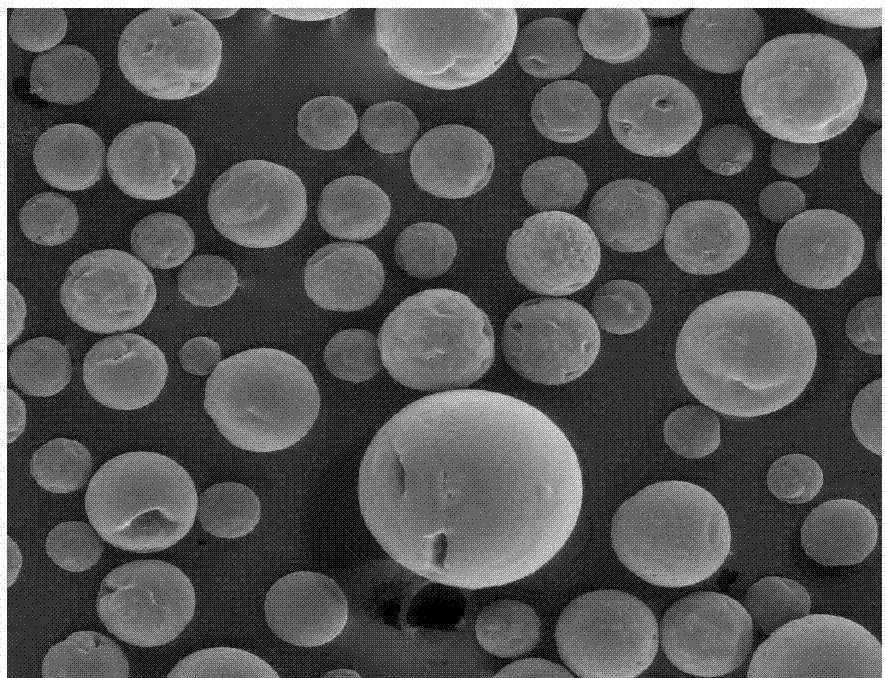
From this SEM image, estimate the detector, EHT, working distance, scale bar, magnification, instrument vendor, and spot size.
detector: LFD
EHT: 10 kV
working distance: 12.9 mm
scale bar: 300000 nm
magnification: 0.2 K X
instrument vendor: FEI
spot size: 4